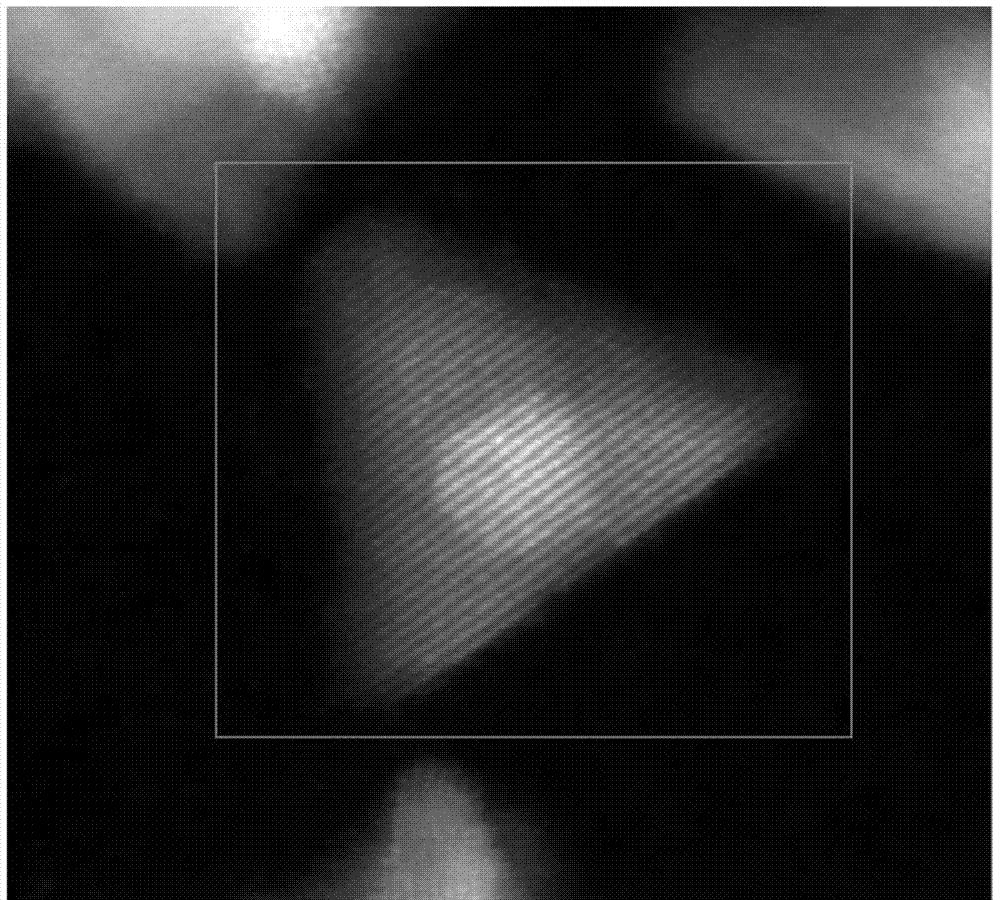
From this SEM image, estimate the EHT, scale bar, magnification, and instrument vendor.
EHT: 200 kV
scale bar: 3 nm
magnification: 5100 K X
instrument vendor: other